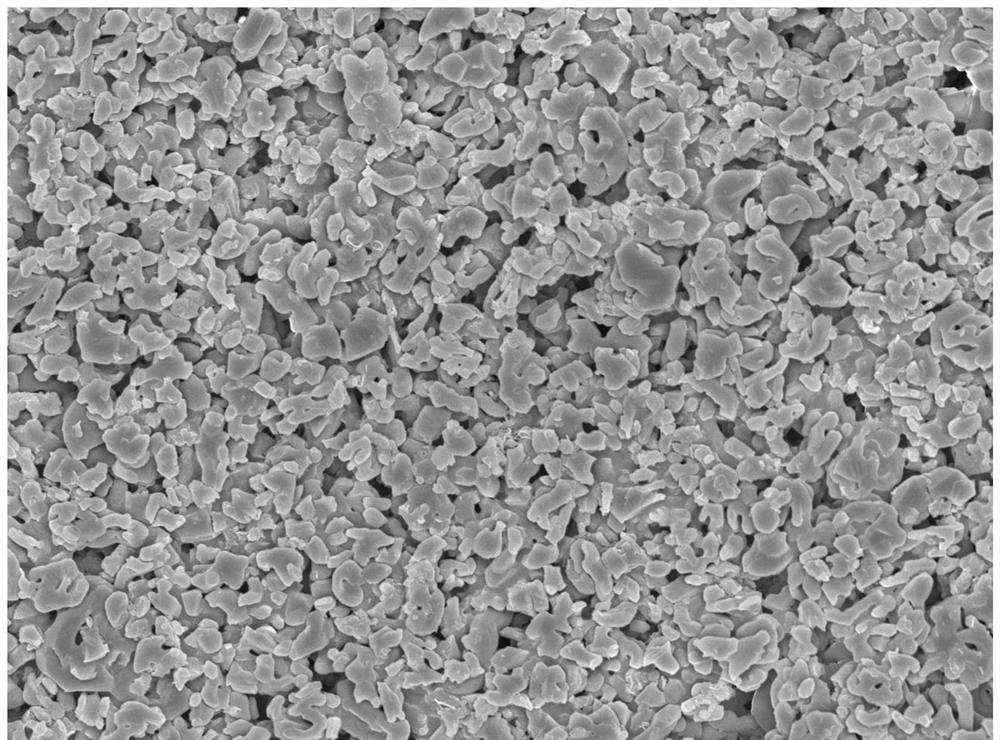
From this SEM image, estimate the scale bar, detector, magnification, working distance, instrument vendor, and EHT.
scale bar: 1000 nm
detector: SEI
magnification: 10 K X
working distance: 8 mm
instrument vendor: JEOL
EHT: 5 kV